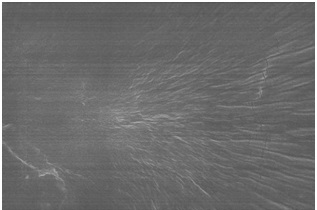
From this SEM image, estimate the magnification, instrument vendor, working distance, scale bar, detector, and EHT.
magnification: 10 K X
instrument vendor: Hitachi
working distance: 6 mm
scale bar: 5000 nm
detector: SE(UL)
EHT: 10 kV